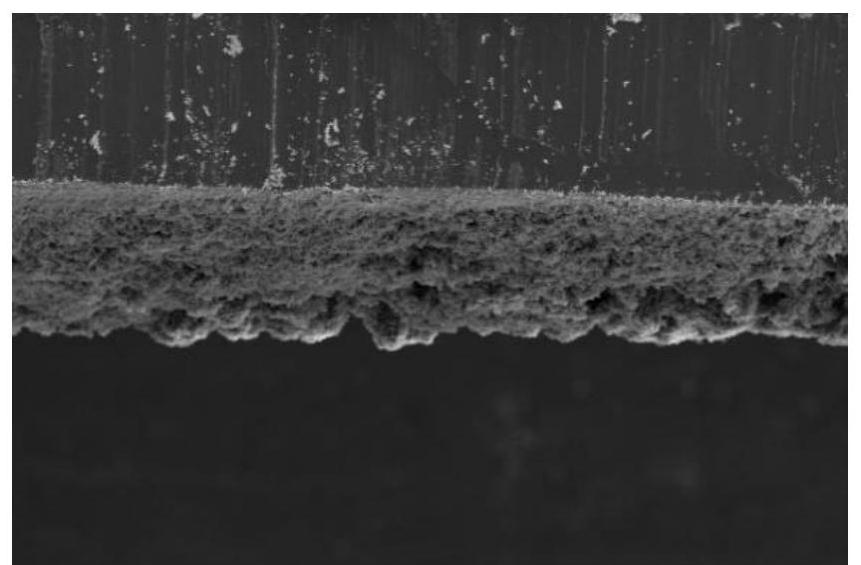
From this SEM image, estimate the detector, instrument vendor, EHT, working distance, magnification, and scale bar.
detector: ETD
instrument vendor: Thermo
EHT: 20 kV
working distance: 12.9 mm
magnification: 0.2 K X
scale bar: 500000 nm